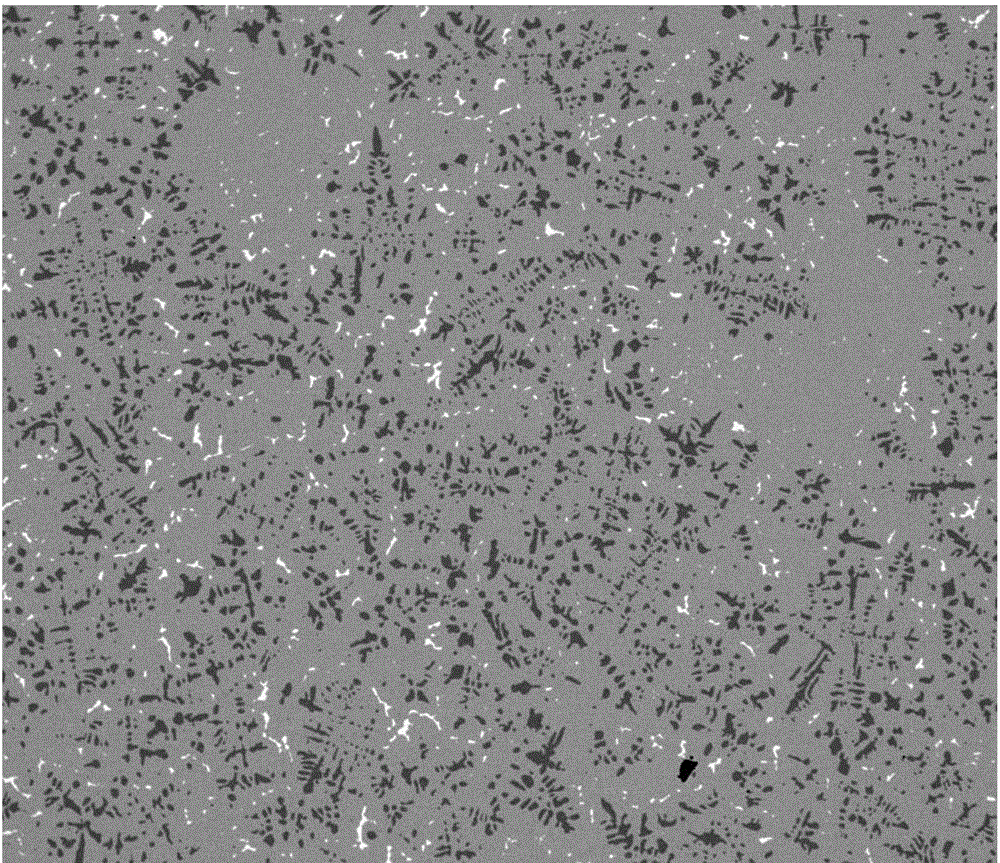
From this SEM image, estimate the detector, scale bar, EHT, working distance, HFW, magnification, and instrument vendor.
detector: vCD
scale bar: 500000 nm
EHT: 20 kV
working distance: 9.5 mm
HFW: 1270 µm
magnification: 0.1 K X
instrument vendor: FEI